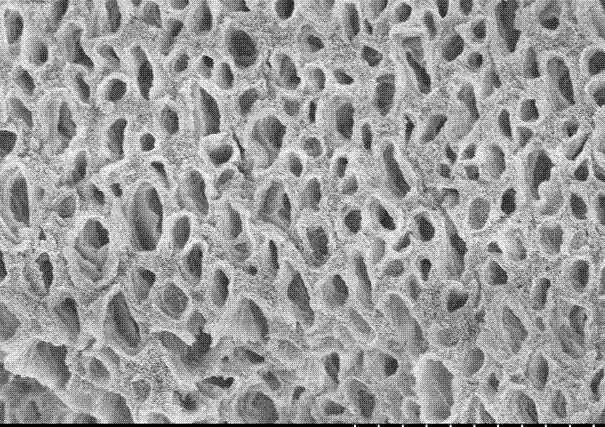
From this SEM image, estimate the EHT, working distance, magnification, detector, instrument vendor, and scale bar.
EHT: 5 kV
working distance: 9.1 mm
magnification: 5 K X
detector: SE(M)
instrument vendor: Hitachi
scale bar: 10000 nm